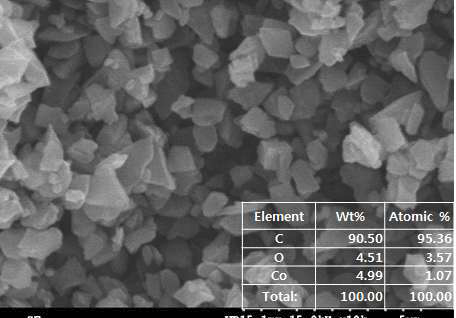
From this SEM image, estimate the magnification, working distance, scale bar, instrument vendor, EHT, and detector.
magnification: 10 K X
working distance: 15.1 mm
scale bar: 5000 nm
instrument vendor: JEOL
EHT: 15 kV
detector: SE